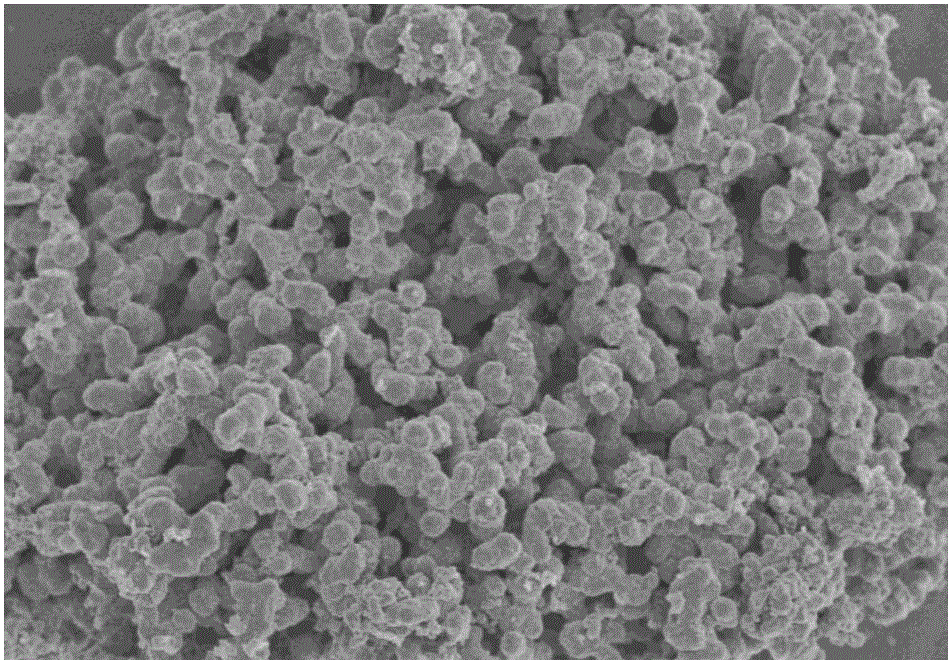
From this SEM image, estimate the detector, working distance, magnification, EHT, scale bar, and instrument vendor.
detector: SE(M)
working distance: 9.4 mm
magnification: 5 K X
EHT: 3 kV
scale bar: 10000 nm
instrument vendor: Hitachi